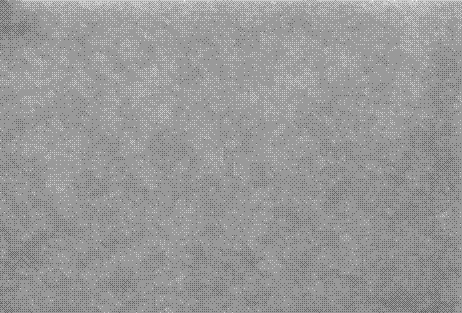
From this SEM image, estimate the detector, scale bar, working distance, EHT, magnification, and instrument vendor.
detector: InLens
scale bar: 200 nm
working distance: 4.1 mm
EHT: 5 kV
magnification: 20 K X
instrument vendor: Zeiss